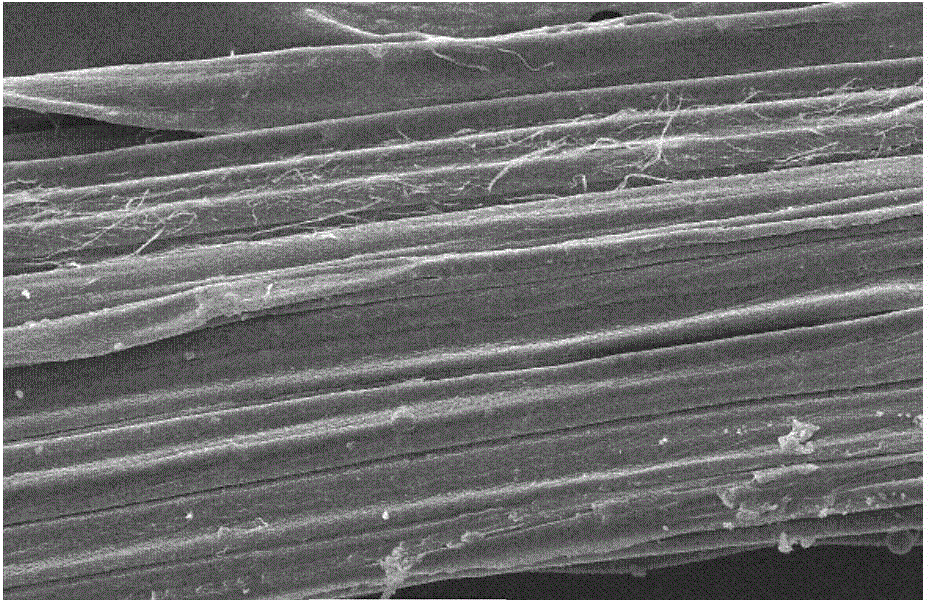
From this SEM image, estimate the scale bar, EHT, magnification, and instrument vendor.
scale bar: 50000 nm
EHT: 15 kV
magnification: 0.5 K X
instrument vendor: JEOL